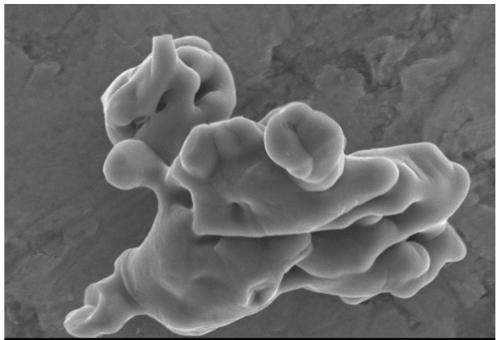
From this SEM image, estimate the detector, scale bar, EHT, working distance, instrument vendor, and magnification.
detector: SEI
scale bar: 2000 nm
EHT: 10 kV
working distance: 15 mm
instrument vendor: JEOL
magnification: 8 K X